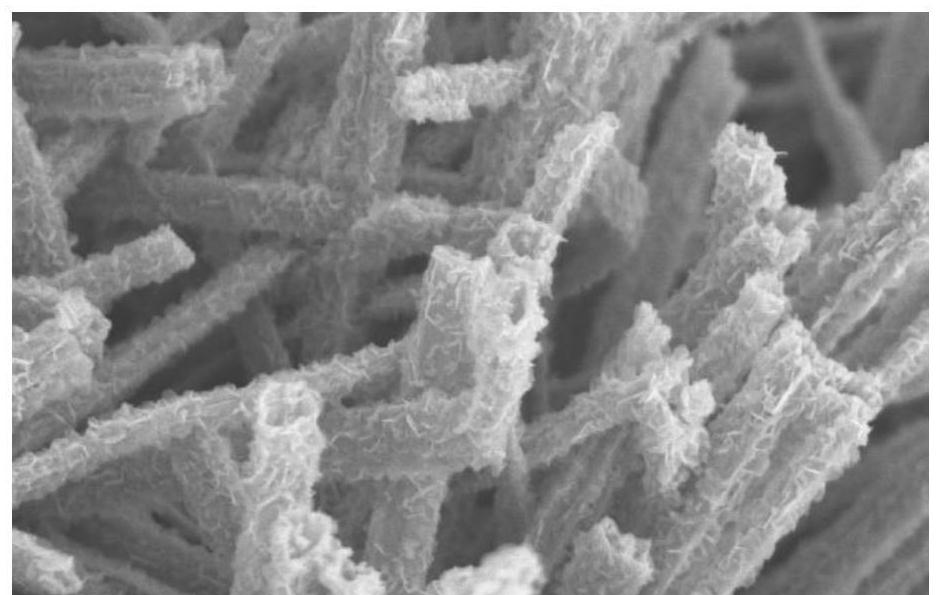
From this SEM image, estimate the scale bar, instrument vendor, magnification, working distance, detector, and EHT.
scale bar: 3000 nm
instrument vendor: Hitachi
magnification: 15 K X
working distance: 9.7 mm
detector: SE(M)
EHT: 5 kV